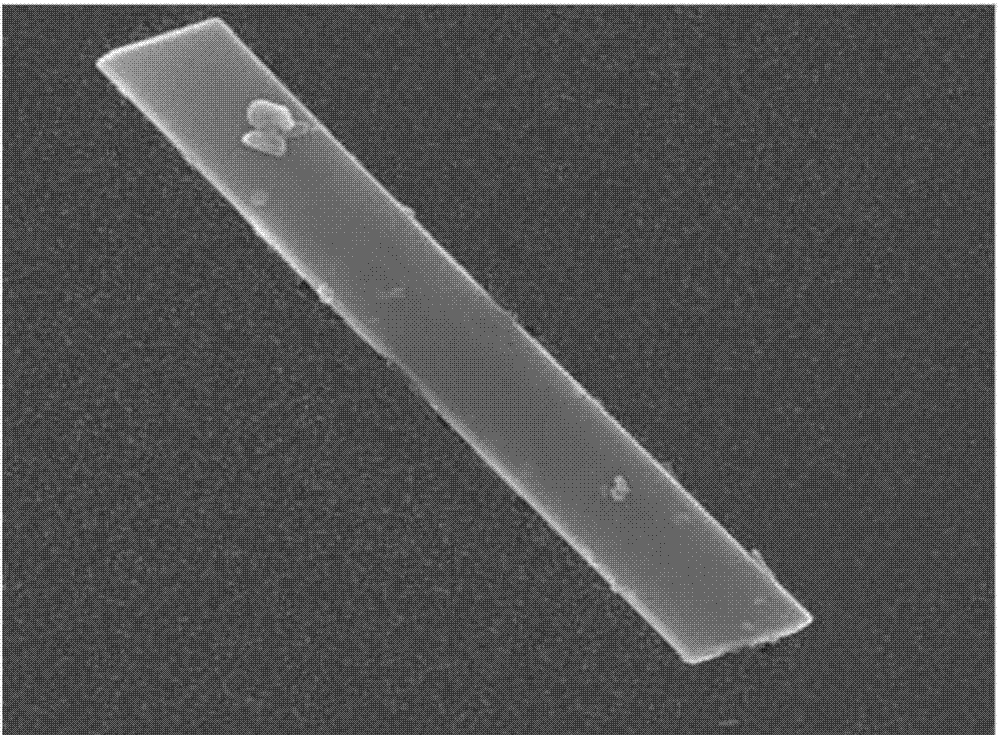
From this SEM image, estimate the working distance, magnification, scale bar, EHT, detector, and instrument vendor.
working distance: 9.3 mm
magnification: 20 K X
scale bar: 1000 nm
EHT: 15 kV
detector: LED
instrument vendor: JEOL